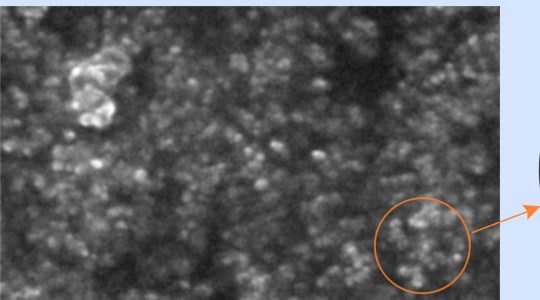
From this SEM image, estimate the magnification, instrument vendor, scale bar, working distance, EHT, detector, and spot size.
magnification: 20 K X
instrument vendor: FEI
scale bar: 1000 nm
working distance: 8 mm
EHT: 20 kV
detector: SE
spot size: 3